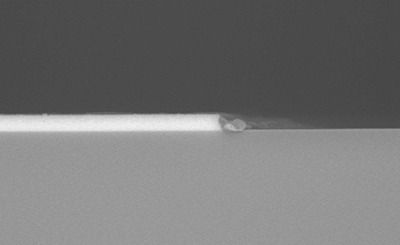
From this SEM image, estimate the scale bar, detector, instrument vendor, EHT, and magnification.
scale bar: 1000 nm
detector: YAGBSE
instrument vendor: Hitachi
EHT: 10 kV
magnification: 30 K X